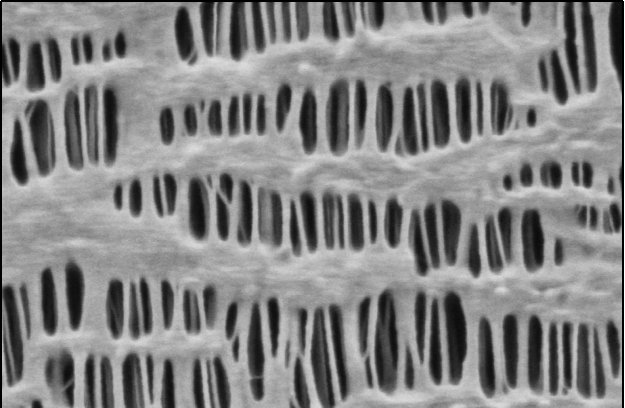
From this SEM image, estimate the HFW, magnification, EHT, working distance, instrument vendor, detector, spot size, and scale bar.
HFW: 2.54 µm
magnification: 50 K X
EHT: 0.2 kV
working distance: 3.1 mm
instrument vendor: Thermo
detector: T1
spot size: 5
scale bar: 500 nm